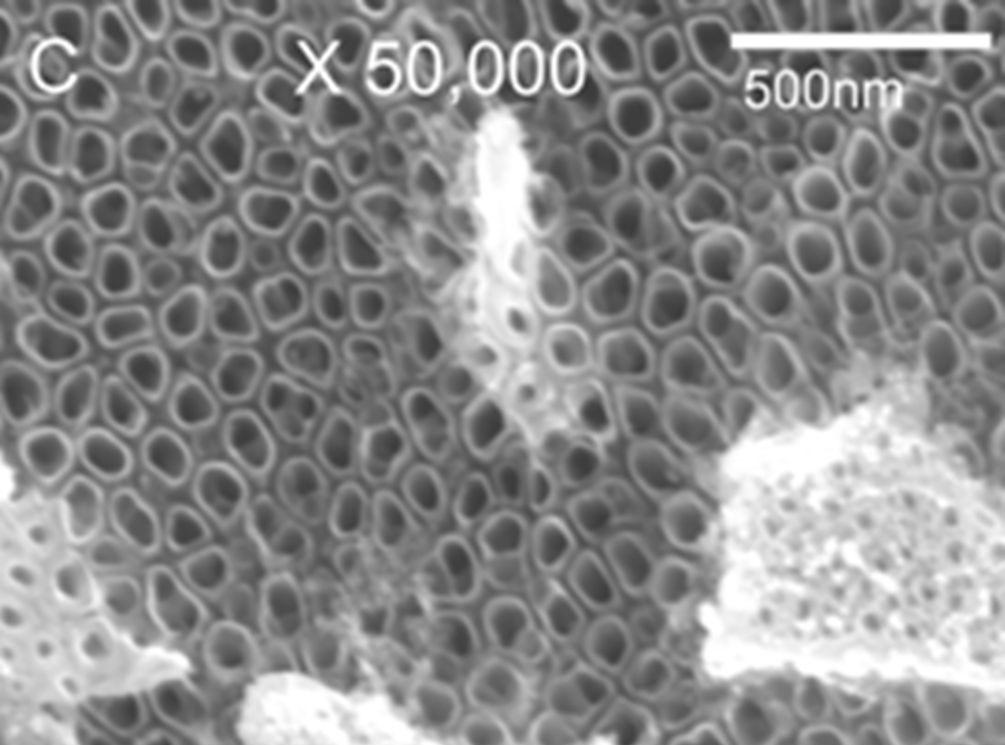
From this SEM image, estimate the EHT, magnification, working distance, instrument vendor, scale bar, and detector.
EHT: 12 kV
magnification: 50 K X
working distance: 10.2 mm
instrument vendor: JEOL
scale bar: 100 nm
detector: SEI